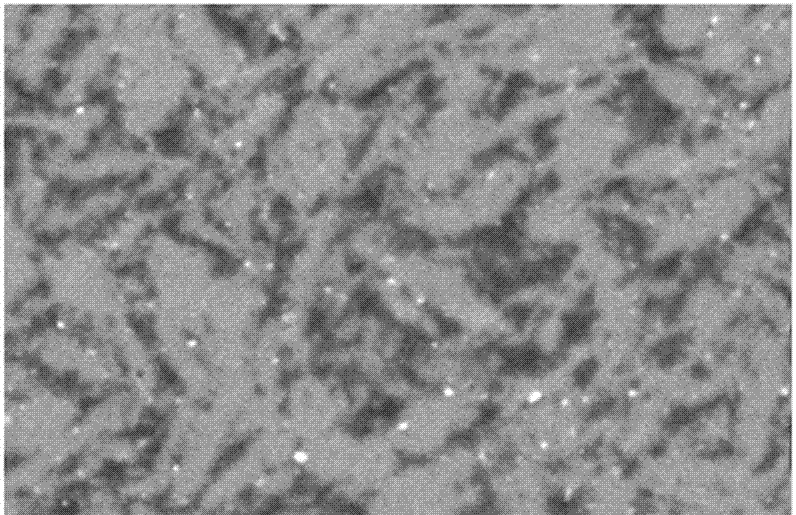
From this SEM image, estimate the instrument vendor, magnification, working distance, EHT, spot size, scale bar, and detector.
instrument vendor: FEI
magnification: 1 K X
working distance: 9.5 mm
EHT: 25 kV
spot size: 5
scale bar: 20000 nm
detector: SE